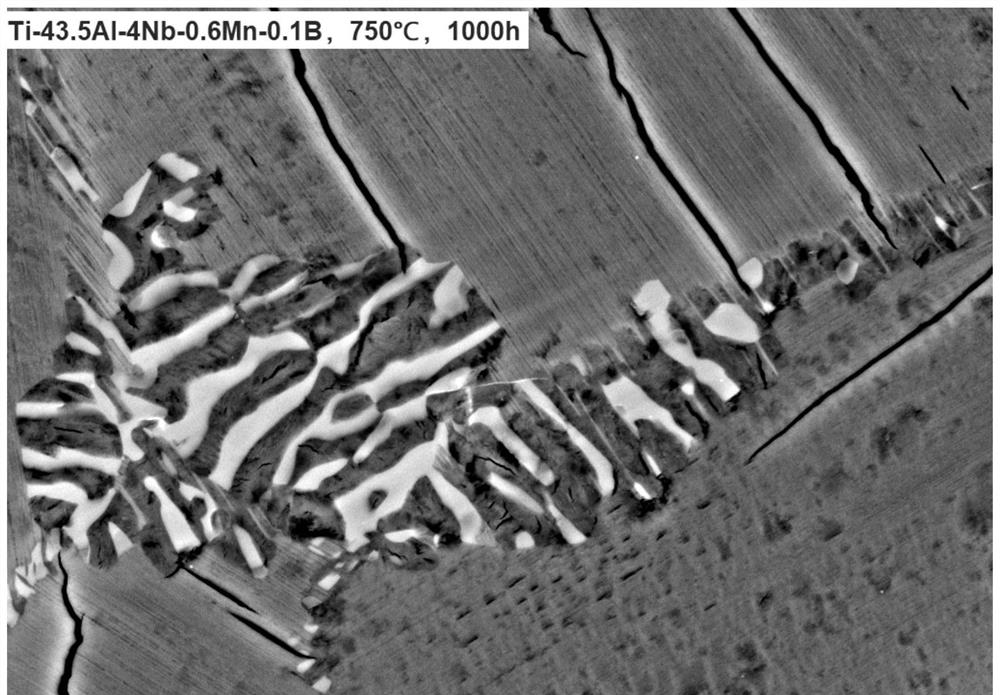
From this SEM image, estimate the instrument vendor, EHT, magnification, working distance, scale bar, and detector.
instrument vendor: Zeiss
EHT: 15 kV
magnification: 3 K X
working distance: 6.4 mm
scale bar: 1000 nm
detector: BSD4 A